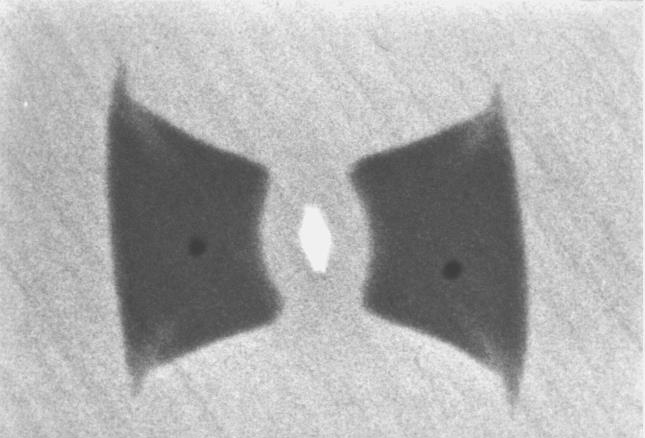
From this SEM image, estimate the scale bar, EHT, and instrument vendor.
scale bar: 10000 nm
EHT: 20 kV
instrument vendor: JEOL